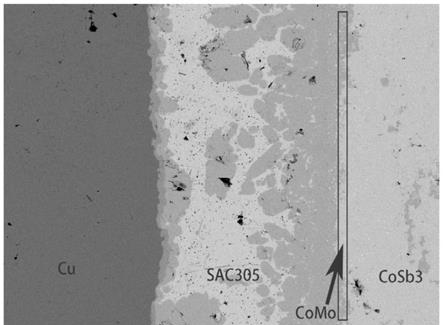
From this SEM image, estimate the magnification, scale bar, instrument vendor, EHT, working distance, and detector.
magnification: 0.3 K X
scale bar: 10000 nm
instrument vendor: JEOL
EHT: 15 kV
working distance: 12 mm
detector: COMPO